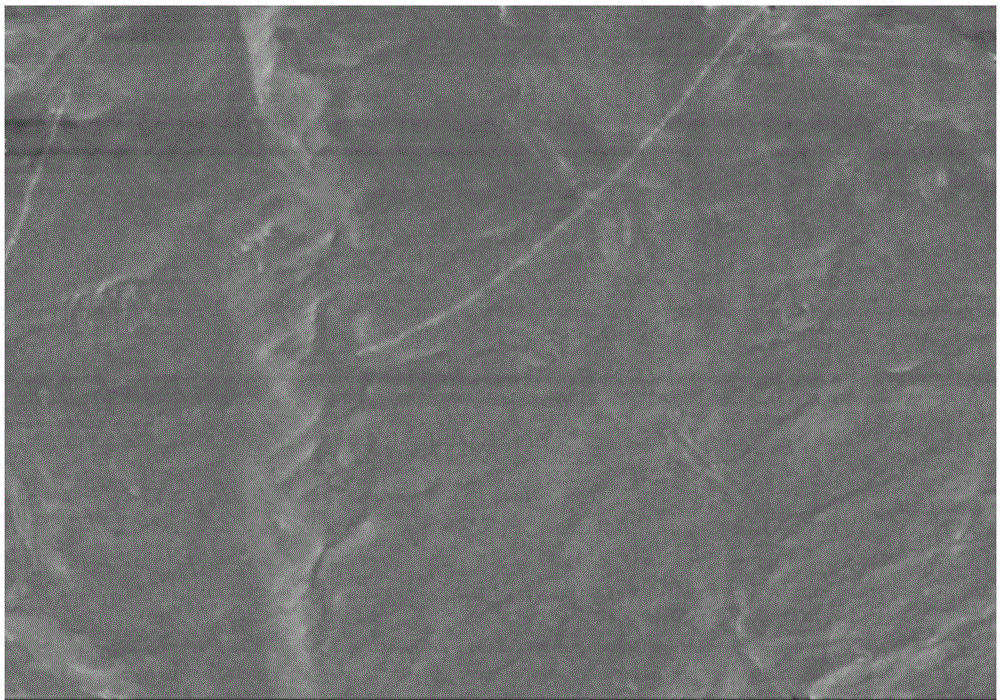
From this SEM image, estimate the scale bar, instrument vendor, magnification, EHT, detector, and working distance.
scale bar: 5000 nm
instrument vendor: Hitachi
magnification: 10 K X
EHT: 5 kV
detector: SE(M)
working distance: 7.9 mm